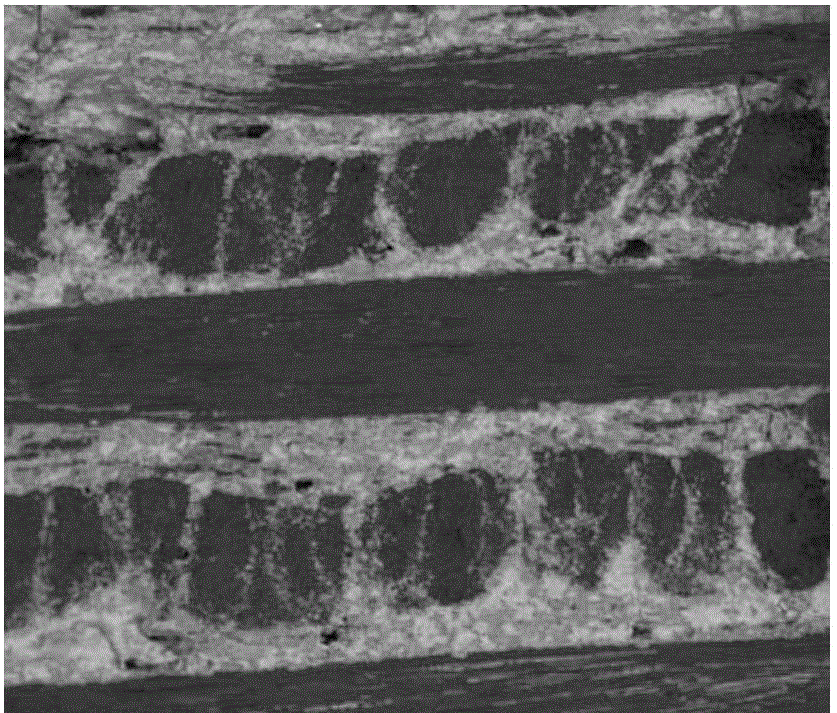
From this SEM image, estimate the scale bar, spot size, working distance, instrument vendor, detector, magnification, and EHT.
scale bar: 500000 nm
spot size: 3.5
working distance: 14.7 mm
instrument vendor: FEI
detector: DualBSD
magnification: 0.05 K X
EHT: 20 kV